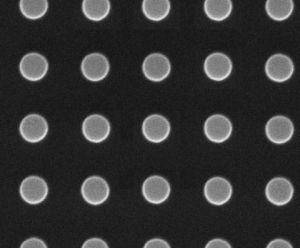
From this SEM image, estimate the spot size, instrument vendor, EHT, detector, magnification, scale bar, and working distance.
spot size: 1.5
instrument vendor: FEI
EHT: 10 kV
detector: ETD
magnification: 75 K X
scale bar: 1000 nm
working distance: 10.2 mm